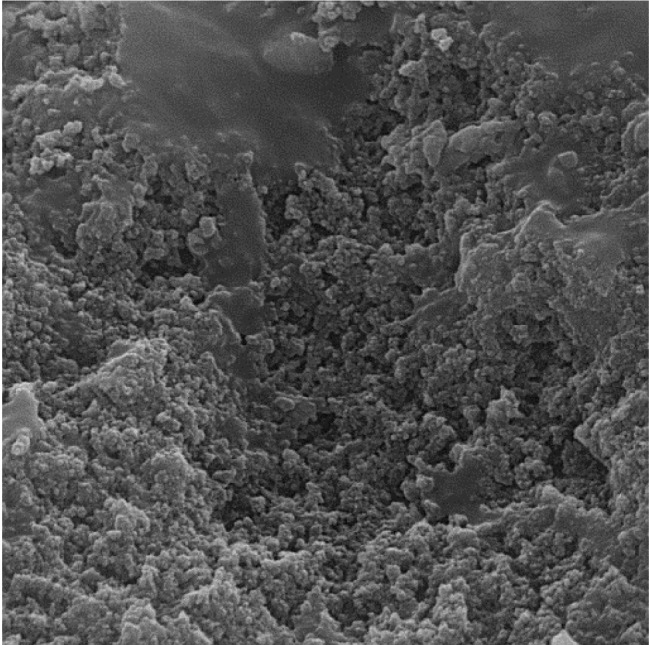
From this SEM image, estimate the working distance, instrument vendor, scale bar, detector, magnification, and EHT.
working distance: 6.891 mm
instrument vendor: Tescan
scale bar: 2000 nm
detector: SE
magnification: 15 K X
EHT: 15 kV